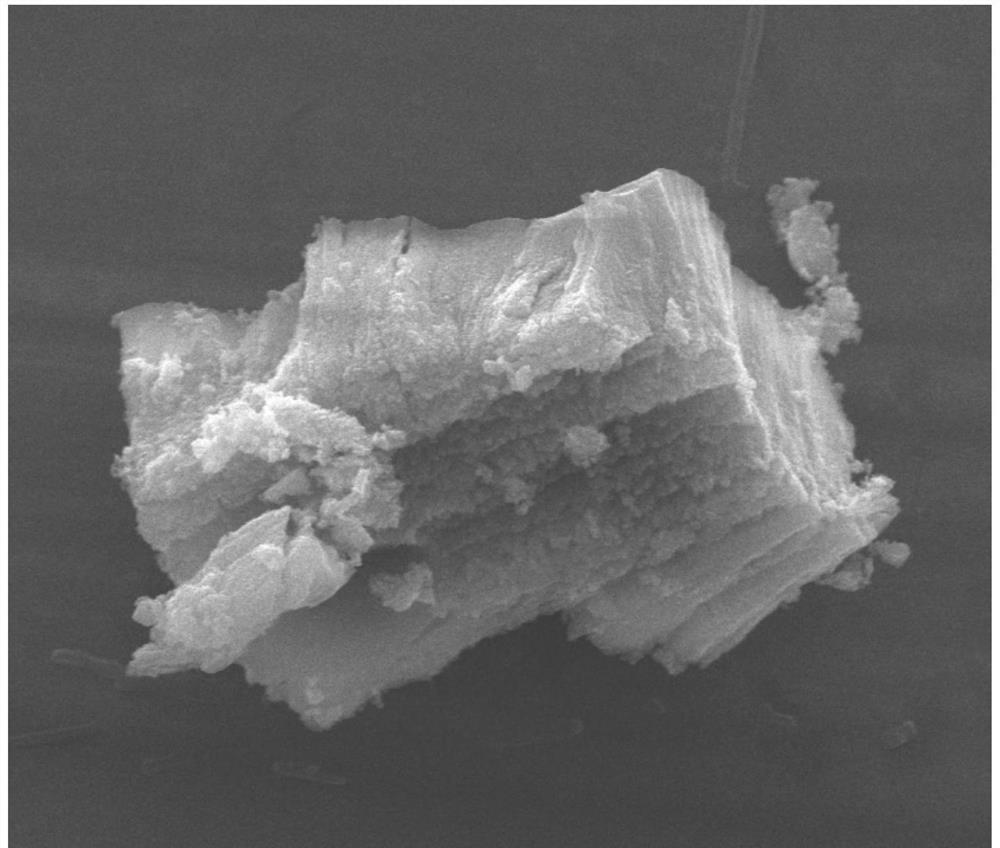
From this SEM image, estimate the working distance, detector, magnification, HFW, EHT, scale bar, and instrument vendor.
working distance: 13 mm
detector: ETD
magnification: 4 K X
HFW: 33.8 µm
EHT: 20 kV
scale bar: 5000 nm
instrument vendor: FEI